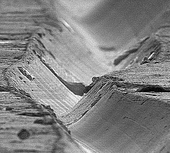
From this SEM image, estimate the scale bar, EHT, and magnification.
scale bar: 20000 nm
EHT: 20 kV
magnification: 0.48 K X